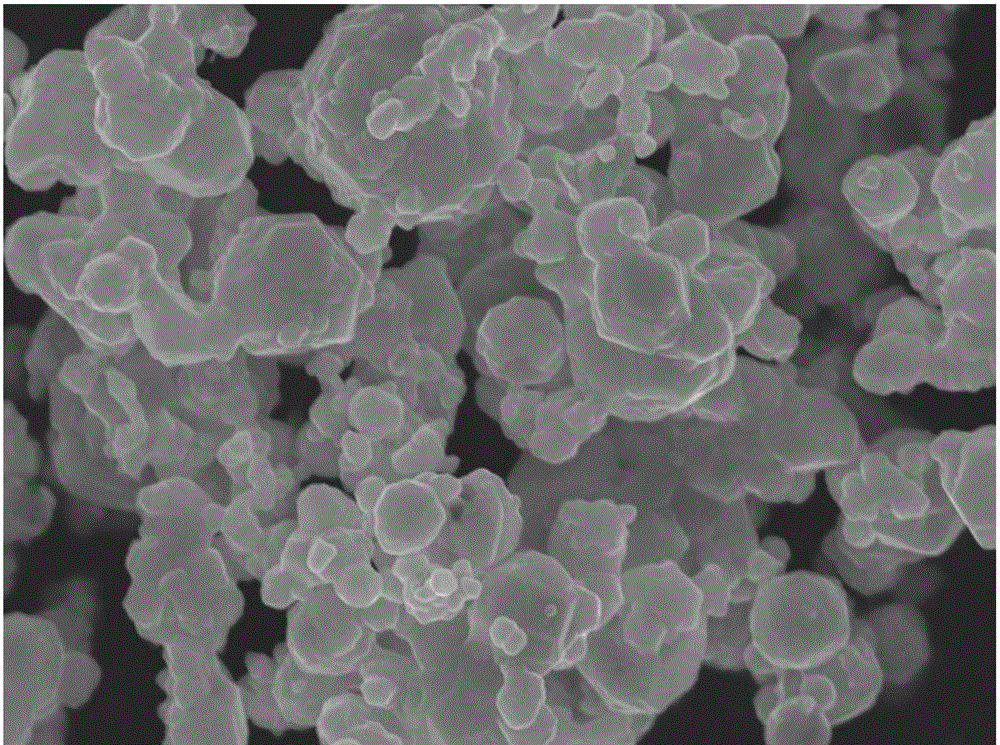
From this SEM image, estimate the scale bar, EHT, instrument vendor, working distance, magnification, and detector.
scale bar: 1000 nm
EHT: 15 kV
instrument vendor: JEOL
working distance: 7.9 mm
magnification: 20 K X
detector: SEI